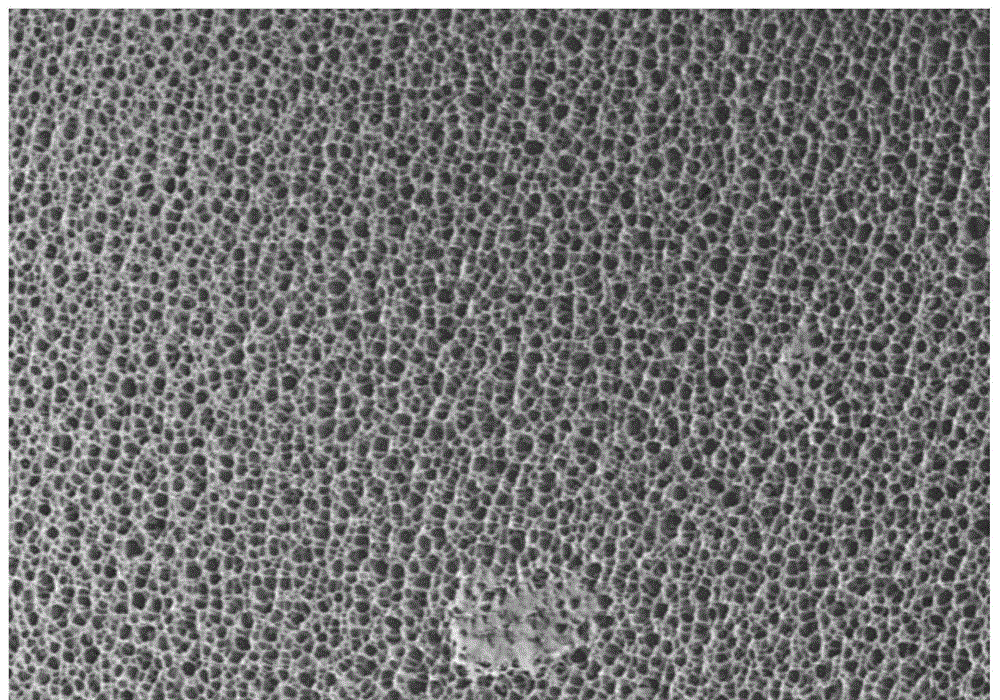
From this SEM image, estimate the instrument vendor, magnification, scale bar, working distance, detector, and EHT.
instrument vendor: Hitachi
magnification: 1 K X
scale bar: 50000 nm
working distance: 14 mm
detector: SE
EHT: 10 kV